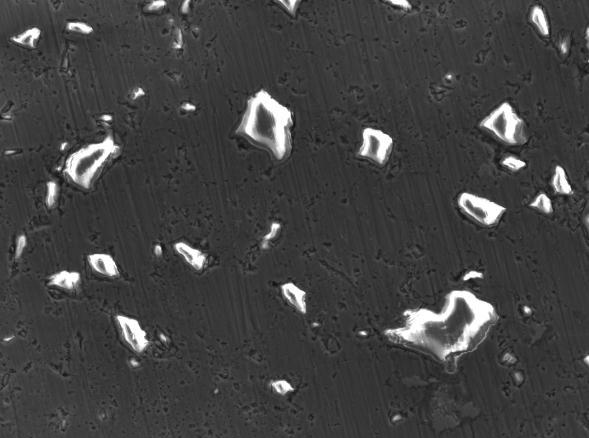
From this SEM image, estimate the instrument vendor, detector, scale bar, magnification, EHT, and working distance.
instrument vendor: JEOL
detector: LED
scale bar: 10000 nm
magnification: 0.33 K X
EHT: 20 kV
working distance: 10.8 mm